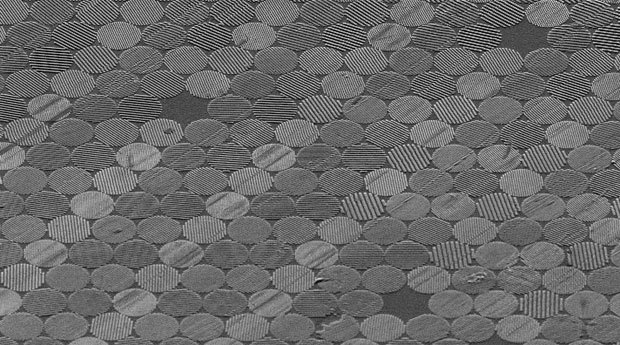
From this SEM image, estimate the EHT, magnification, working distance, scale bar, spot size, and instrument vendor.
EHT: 5 kV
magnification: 2 K X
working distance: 13 mm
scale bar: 20000 nm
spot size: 3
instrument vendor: FEI